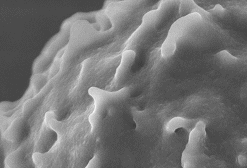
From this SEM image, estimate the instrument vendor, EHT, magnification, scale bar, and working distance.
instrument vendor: Hitachi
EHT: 3 kV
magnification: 50 K X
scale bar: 1000 nm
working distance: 8 mm